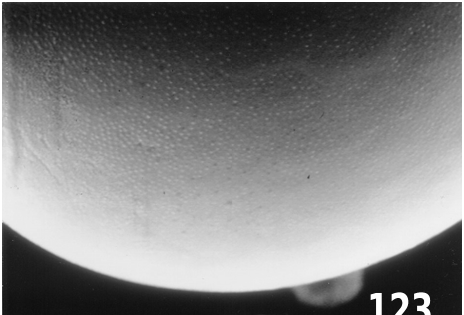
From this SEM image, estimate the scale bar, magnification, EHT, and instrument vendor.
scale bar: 10000 nm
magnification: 1 K X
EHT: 10 kV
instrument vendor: other